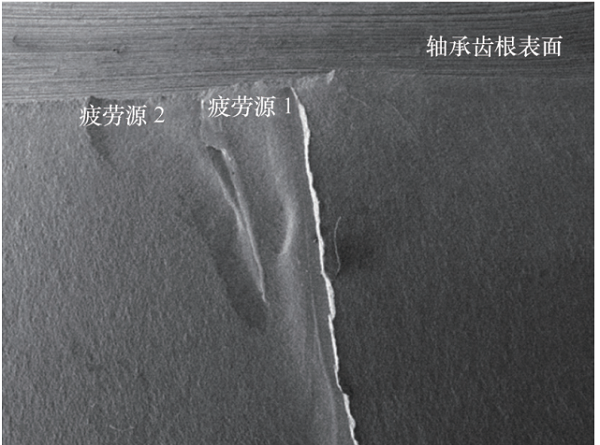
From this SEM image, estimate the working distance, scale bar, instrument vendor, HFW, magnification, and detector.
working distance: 13.47 mm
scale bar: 2000000 nm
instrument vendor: Tescan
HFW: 12000 µm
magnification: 0.023 K X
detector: SE